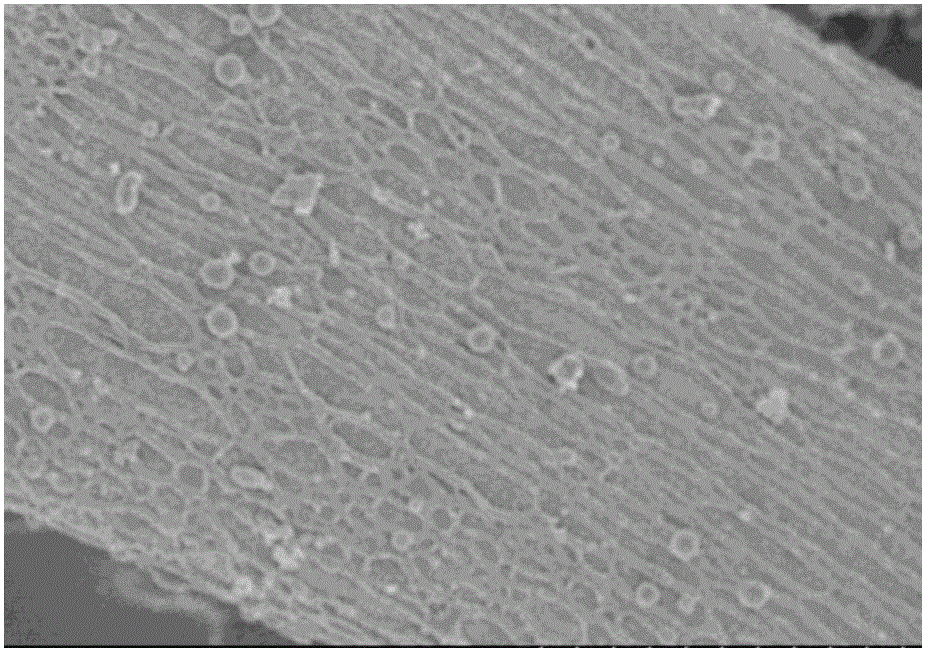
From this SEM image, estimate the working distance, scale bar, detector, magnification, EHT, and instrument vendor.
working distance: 9.4 mm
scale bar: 10000 nm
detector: SE(M)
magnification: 5 K X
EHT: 3 kV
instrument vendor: Hitachi